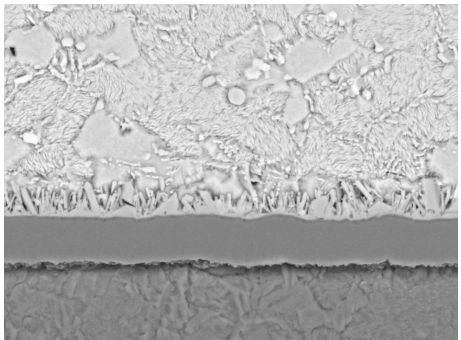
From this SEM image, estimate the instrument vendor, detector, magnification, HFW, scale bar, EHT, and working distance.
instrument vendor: Tescan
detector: BSE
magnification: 6.03 K X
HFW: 45.9 µm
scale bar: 10000 nm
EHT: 20 kV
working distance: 14.93 mm